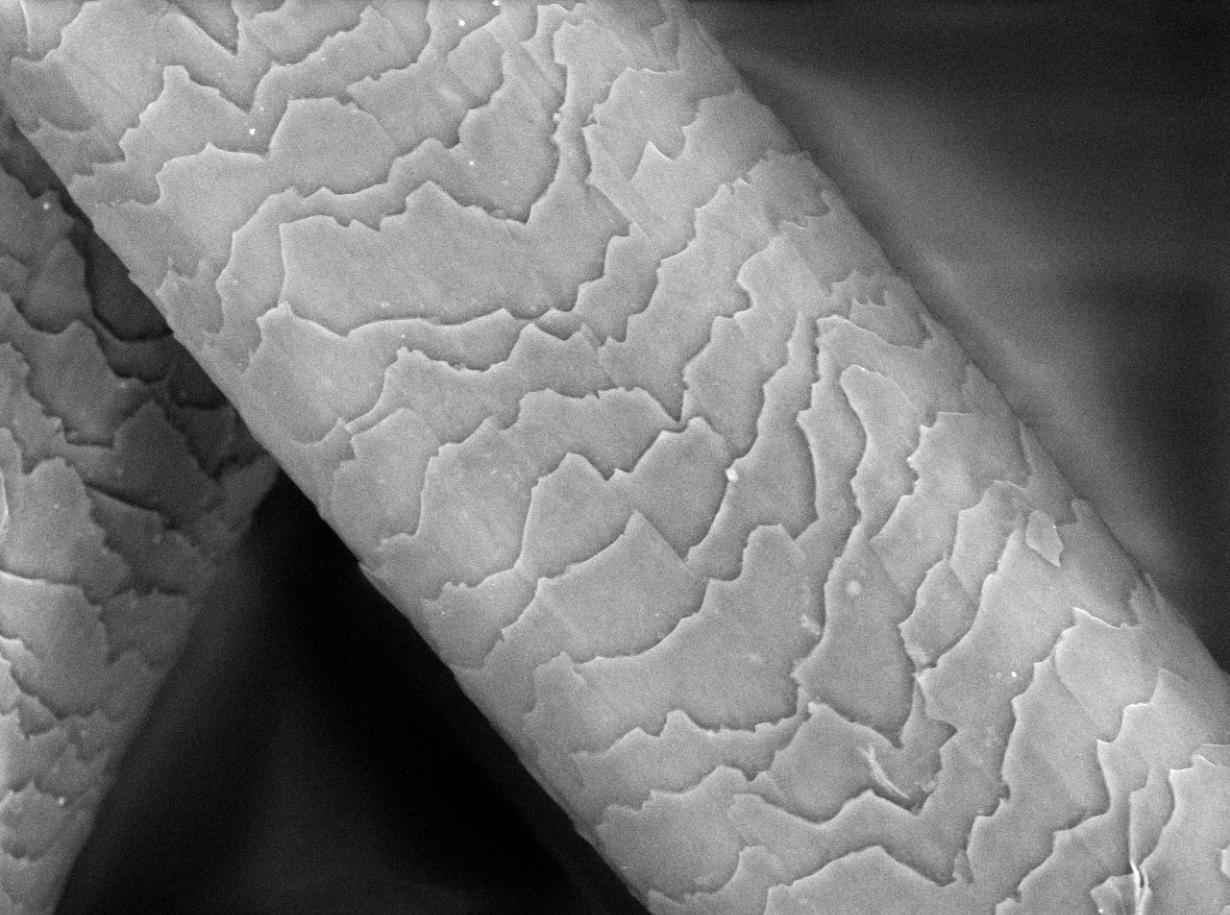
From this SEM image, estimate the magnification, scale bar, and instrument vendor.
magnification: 1.5 K X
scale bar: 50000 nm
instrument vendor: Hitachi